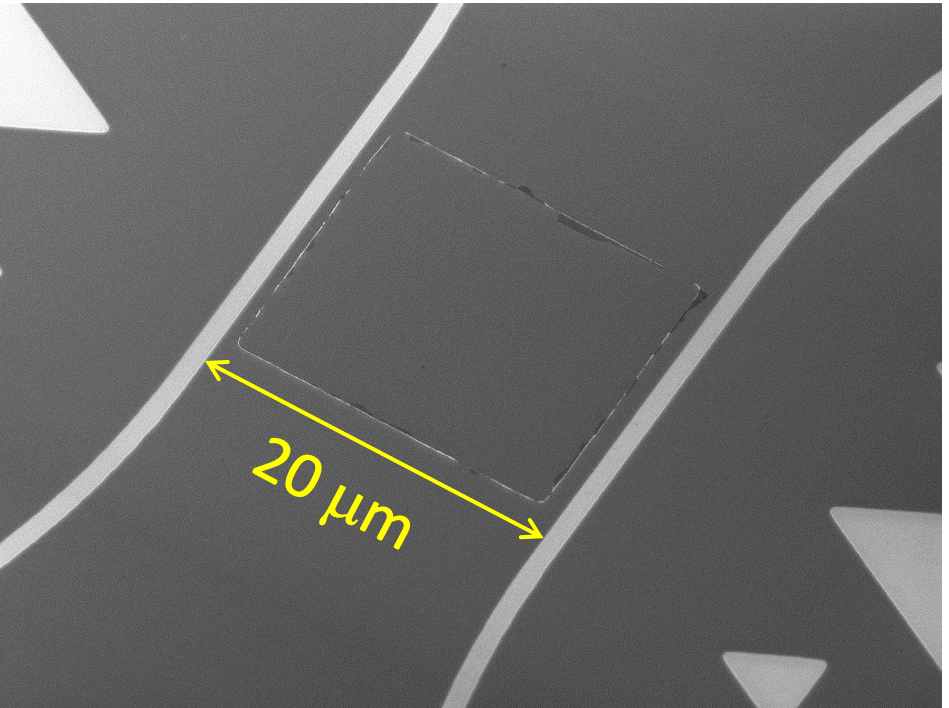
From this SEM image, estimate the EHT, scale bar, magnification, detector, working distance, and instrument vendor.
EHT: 5 kV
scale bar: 10000 nm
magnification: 2.5 K X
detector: SEI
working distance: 8.1 mm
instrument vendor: JEOL